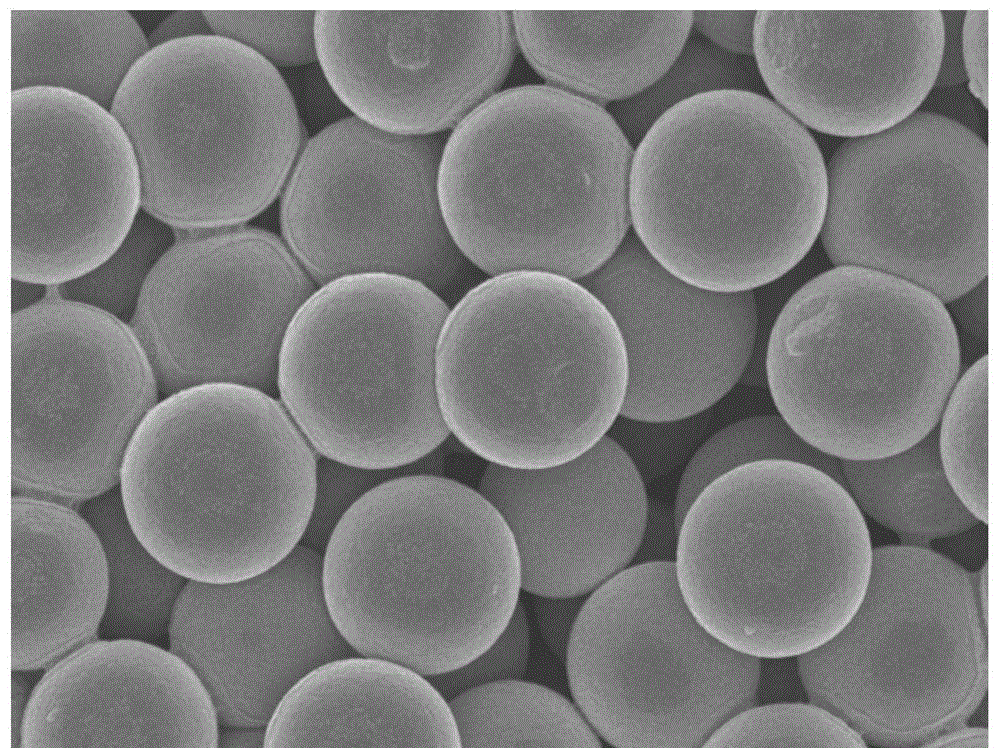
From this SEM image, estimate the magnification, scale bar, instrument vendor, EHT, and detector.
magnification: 30 K X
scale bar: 100 nm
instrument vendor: JEOL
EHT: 5 kV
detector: SEI